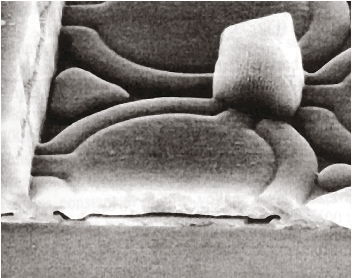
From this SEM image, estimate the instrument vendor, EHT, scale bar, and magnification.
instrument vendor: JEOL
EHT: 15 kV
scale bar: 3750 nm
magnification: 8 K X